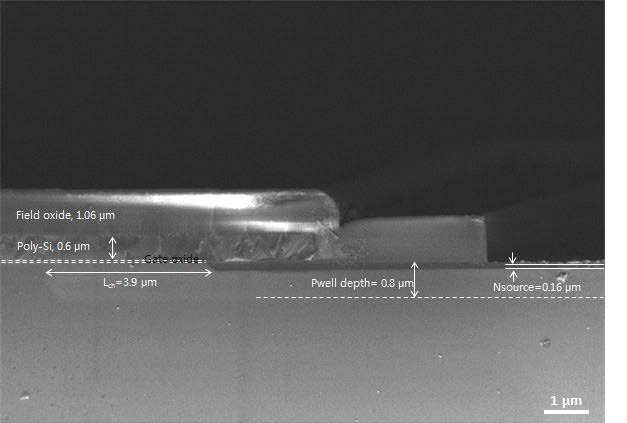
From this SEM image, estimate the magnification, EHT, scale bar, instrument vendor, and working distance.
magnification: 20 K X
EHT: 2 kV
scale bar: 1000 nm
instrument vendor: Zeiss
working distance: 3.4 mm